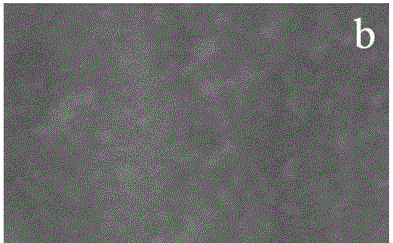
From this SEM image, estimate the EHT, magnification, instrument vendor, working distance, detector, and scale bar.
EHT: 5 kV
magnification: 100 K X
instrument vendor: Hitachi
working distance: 8.7 mm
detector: SE(M,LA0)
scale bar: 500 nm